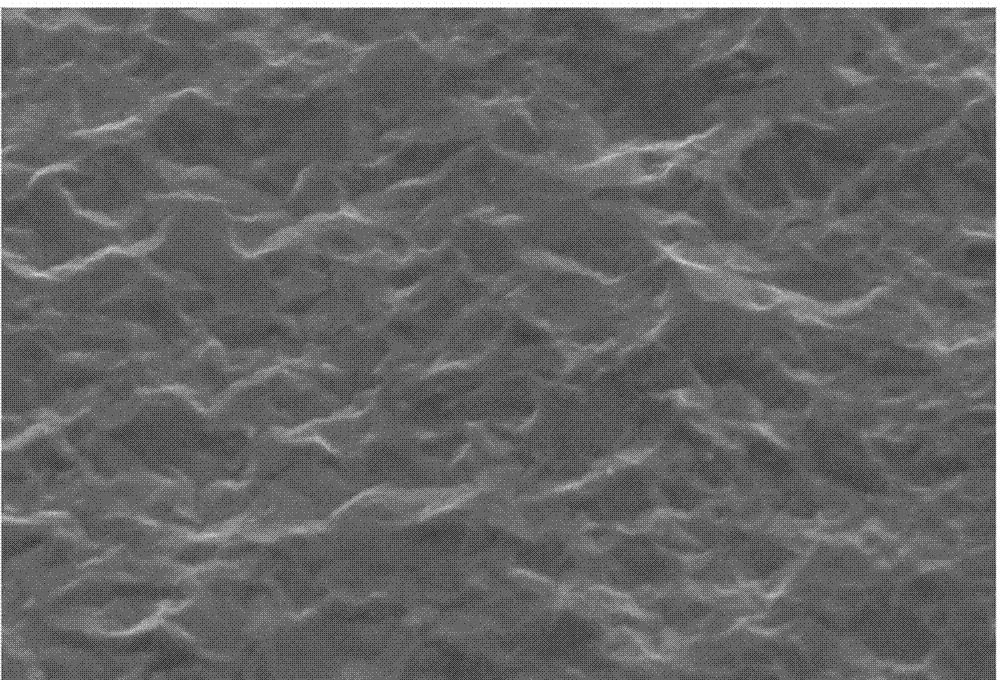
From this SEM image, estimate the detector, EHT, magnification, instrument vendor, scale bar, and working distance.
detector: SE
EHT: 15 kV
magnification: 3 K X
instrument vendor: Hitachi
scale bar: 10000 nm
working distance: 19.5 mm